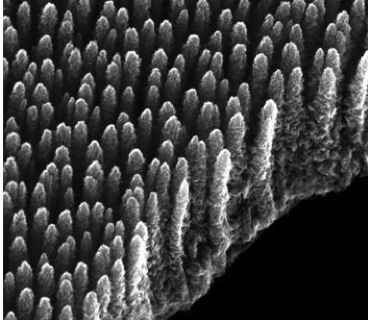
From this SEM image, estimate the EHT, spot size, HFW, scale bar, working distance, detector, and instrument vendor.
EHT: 30 kV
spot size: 1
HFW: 2.91 µm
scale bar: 1000 nm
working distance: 8.6 mm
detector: ETD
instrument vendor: FEI